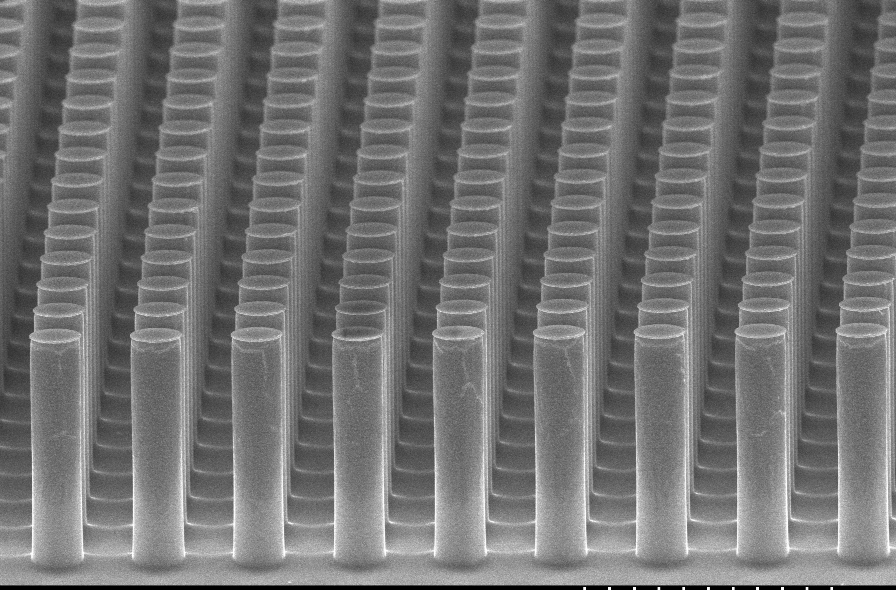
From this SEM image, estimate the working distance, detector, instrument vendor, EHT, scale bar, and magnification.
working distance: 25.2 mm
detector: SE(U)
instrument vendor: Hitachi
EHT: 10 kV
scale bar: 10000 nm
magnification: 3.5 K X